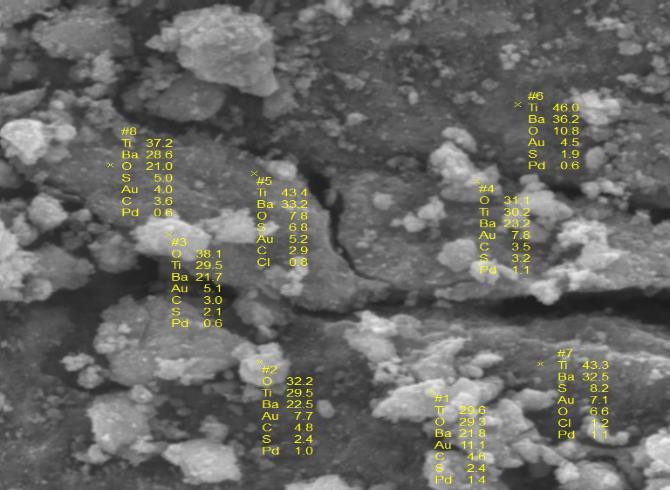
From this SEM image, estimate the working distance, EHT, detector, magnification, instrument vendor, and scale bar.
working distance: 15 mm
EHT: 20 kV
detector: SE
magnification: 3 K X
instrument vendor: Tescan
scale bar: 10000 nm